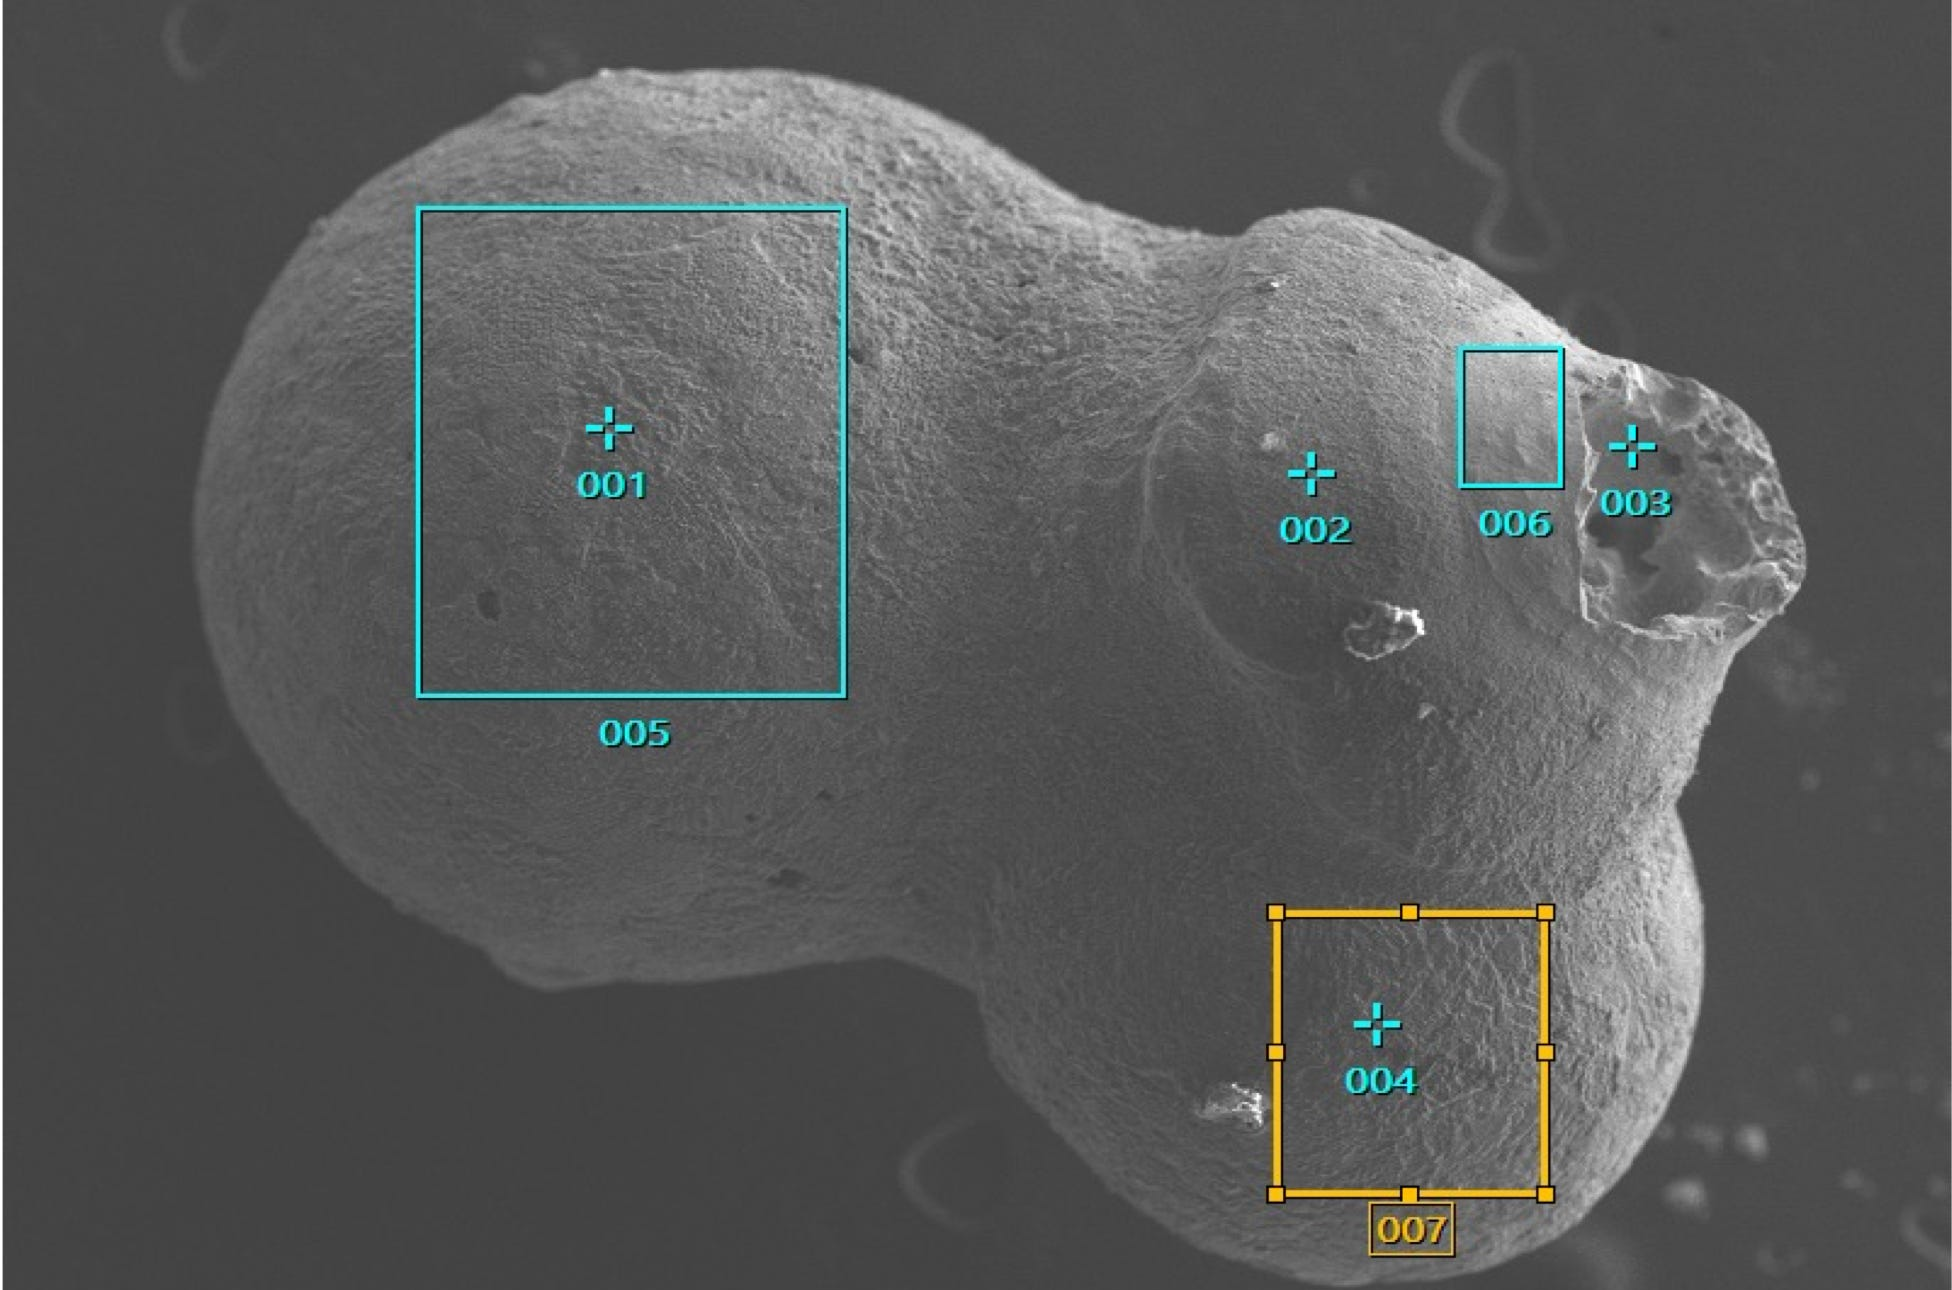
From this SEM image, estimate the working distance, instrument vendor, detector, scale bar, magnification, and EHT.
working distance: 11.1 mm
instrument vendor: JEOL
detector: SEI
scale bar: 100000 nm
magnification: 0.07 K X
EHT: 15 kV